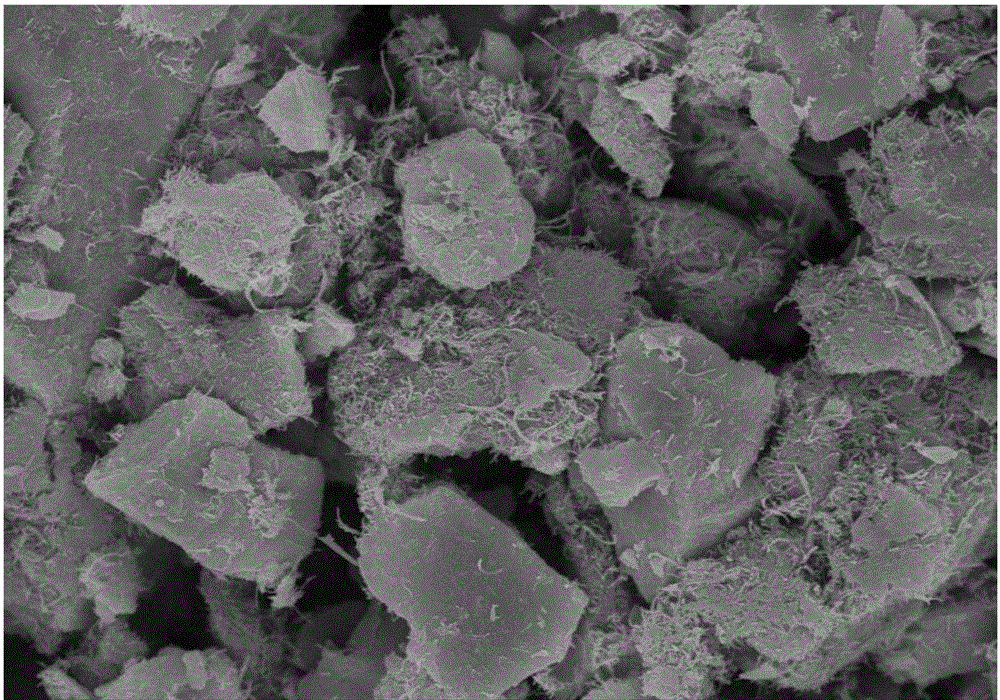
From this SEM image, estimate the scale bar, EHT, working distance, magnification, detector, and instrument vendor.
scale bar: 10000 nm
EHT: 15 kV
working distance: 7.6 mm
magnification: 5 K X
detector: SE(M)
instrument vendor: Hitachi